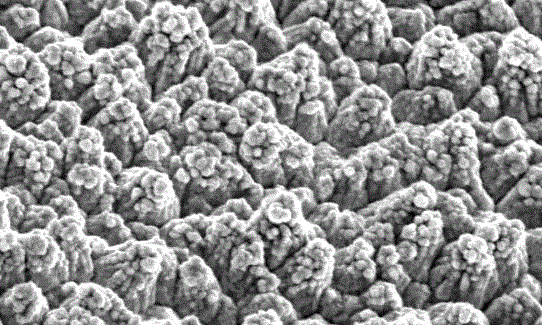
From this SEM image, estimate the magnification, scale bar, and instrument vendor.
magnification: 1 K X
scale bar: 10000 nm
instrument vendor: Hitachi